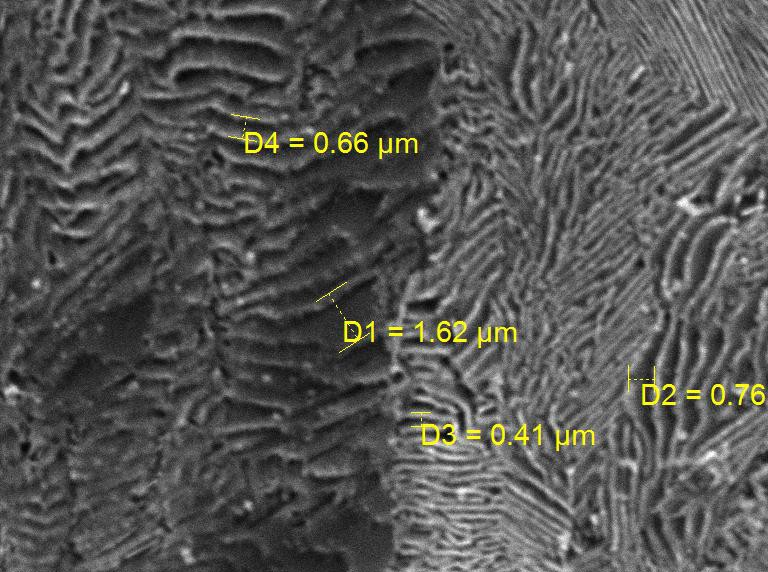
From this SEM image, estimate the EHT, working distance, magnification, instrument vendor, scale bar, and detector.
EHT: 30 kV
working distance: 15.27 mm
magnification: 10 K X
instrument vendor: Tescan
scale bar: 5000 nm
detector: SE